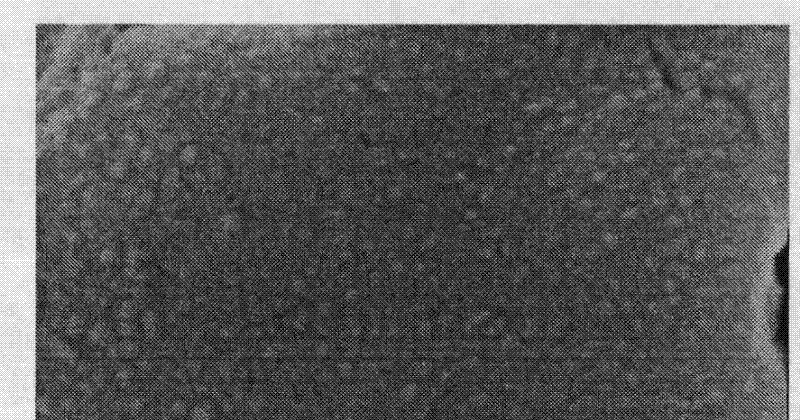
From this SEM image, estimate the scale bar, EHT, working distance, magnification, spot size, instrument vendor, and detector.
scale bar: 200 nm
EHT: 20 kV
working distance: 9.5 mm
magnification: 120 K X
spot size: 3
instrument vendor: FEI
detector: SE